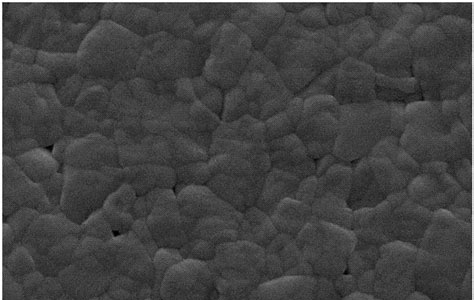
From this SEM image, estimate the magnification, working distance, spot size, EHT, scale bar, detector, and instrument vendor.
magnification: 20 K X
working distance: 6.1 mm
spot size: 3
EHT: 20 kV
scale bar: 1000 nm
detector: SE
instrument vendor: FEI